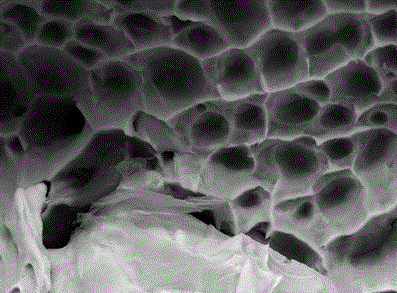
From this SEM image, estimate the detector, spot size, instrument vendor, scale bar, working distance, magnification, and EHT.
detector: SE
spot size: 4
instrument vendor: FEI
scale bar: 5000 nm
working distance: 5.7 mm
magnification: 4 K X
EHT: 20 kV